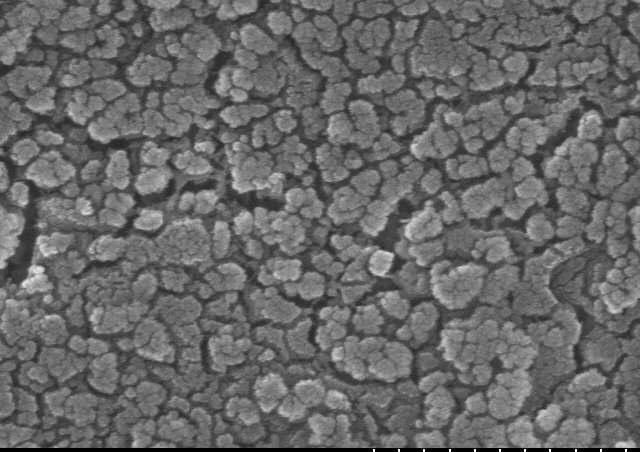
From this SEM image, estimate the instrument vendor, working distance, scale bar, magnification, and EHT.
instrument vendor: Hitachi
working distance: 11.6 mm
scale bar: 500 nm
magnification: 100 K X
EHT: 15 kV